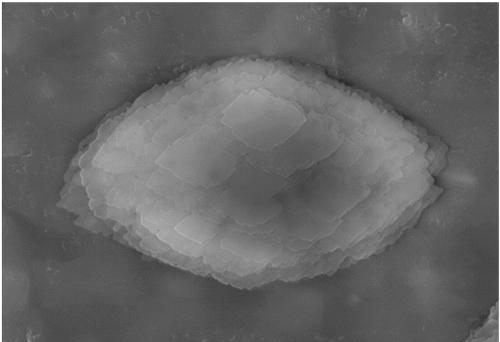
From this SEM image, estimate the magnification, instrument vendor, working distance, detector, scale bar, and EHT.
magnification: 10 K X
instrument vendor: Hitachi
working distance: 8.7 mm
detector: SE(M)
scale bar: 5000 nm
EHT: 15 kV